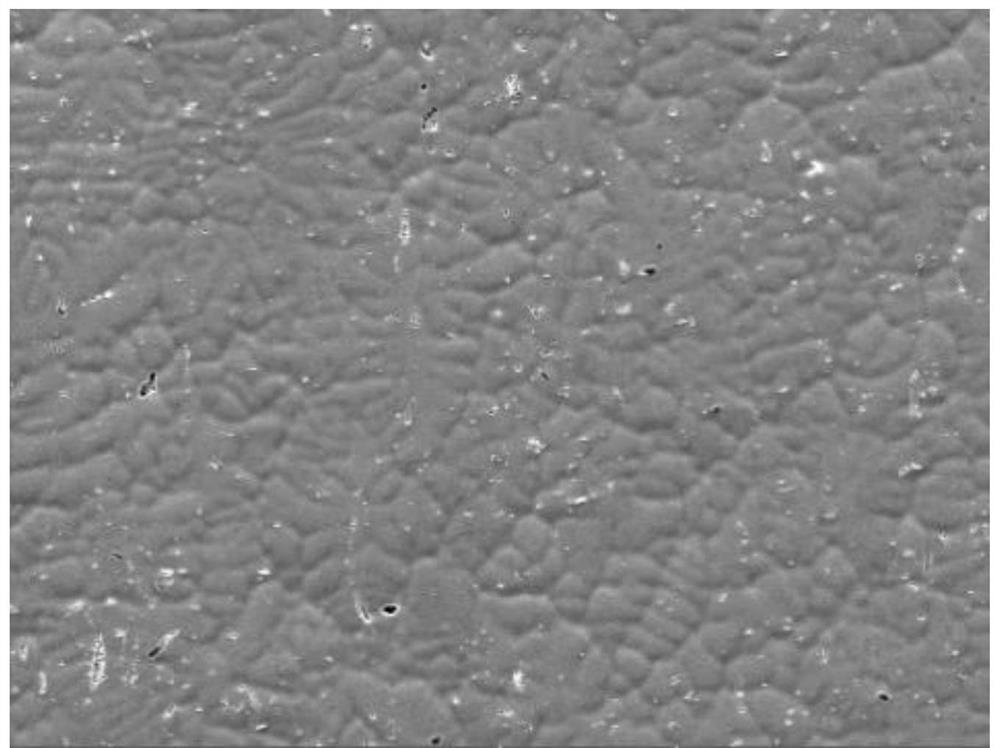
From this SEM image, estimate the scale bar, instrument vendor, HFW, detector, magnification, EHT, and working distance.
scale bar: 100000 nm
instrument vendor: Tescan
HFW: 554 µm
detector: SE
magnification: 0.5 K X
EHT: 20 kV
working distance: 14.94 mm